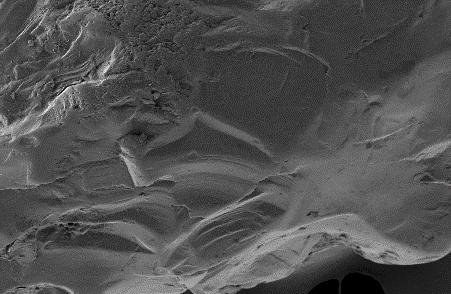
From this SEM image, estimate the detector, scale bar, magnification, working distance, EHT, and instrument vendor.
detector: SE2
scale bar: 100000 nm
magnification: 0.5 K X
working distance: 3.3 mm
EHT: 5 kV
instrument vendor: Zeiss